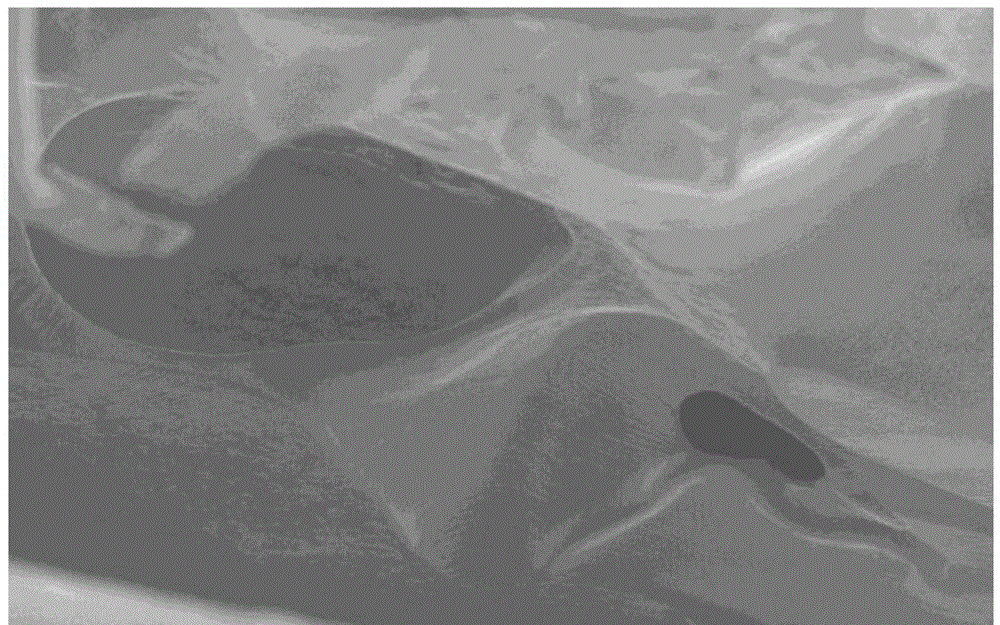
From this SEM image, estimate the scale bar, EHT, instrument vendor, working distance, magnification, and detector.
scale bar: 100000 nm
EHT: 2 kV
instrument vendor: Hitachi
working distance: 7.8 mm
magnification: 0.4 K X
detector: SE(M)